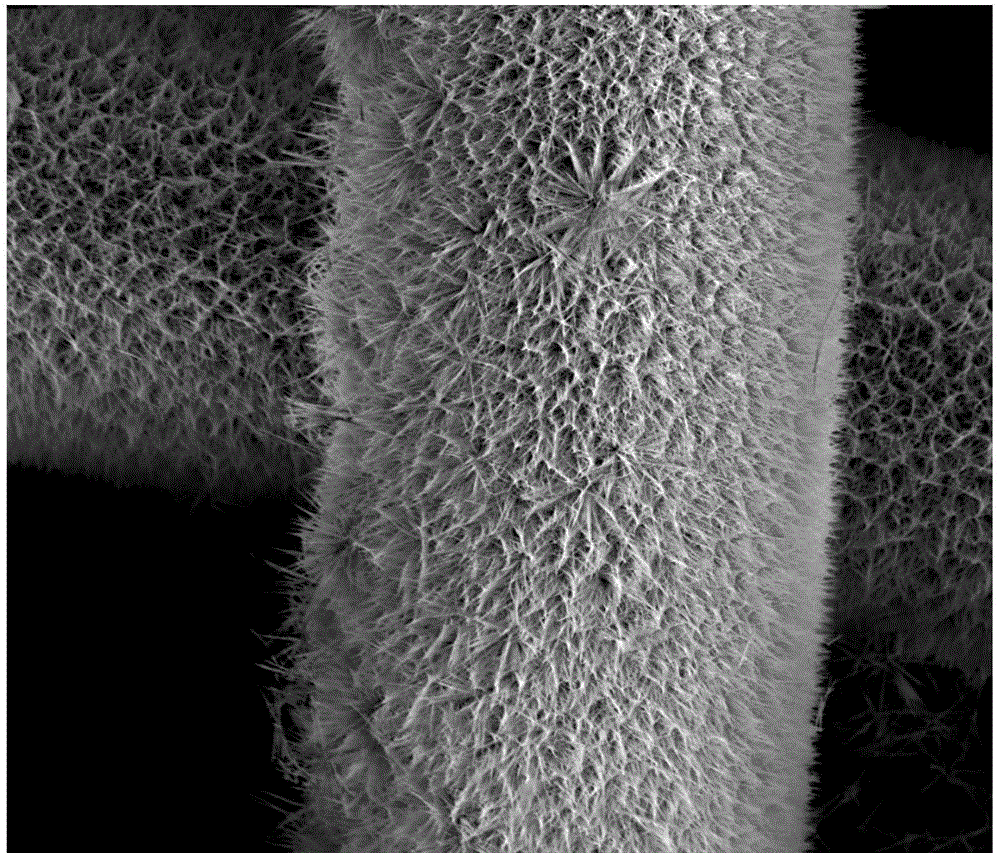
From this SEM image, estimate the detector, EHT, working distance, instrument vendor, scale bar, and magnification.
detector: ETD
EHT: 10 kV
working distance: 5.6 mm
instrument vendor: FEI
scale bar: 50000 nm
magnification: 1.2 K X